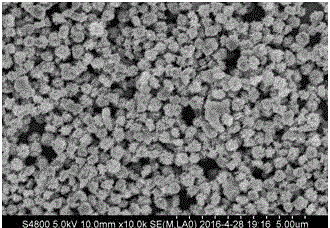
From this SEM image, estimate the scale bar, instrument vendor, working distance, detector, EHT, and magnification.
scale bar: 5000 nm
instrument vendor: Hitachi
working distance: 10 mm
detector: SE(M,LA0)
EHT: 5 kV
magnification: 10 K X